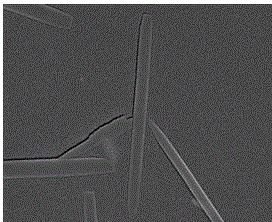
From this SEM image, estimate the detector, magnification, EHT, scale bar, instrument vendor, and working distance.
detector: InLens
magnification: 30 K X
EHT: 5 kV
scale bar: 200 nm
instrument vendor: Zeiss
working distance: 4.7 mm